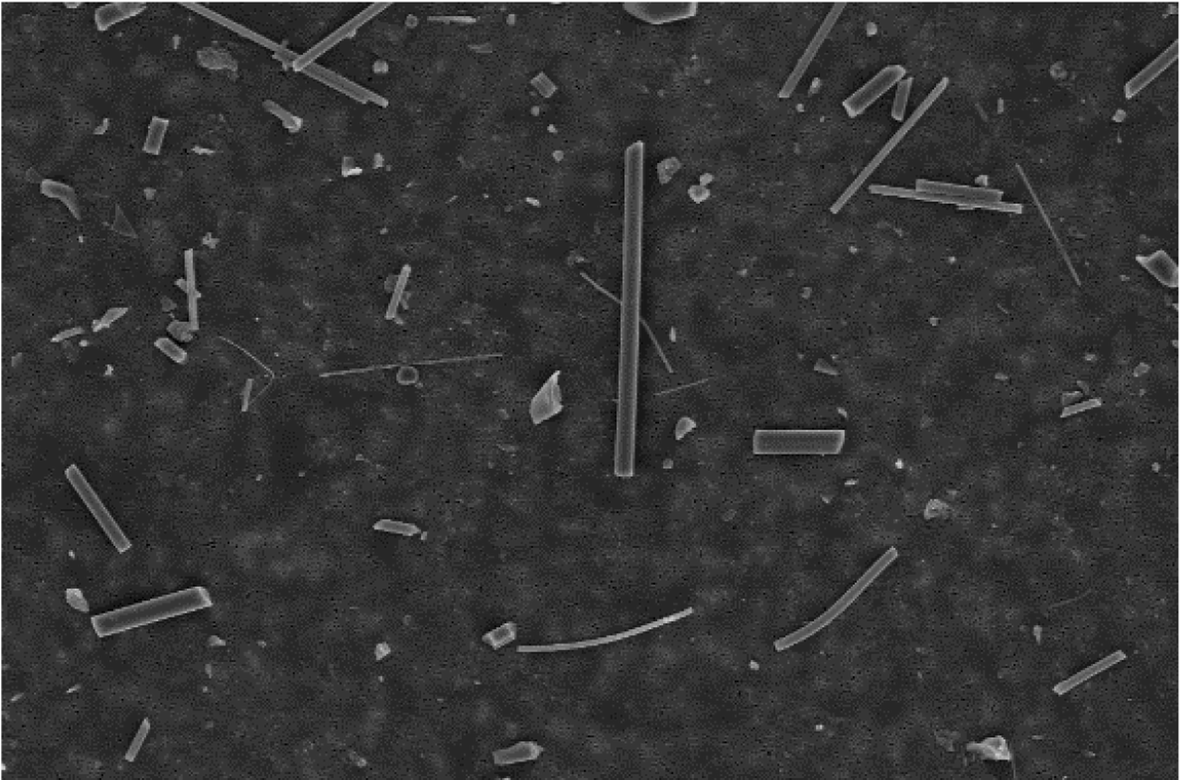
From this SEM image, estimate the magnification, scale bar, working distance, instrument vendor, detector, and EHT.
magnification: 1 K X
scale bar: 100000 nm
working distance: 31 mm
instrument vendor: Zeiss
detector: SE1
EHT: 20 kV